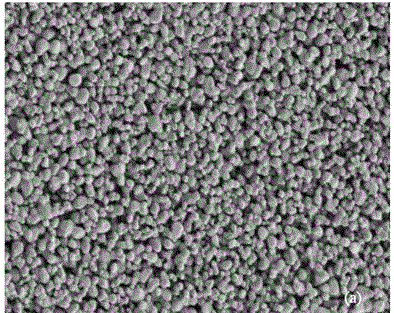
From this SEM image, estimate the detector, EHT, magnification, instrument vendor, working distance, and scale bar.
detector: SE(M)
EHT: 1 kV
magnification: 15 K X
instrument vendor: Hitachi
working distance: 14.3 mm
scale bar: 3000 nm